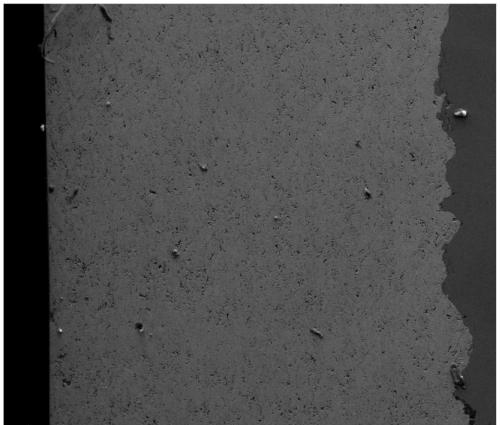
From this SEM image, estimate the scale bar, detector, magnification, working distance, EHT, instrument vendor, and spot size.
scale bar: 300000 nm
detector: ETD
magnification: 0.25 K X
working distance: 7.8 mm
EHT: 20 kV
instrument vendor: FEI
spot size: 6